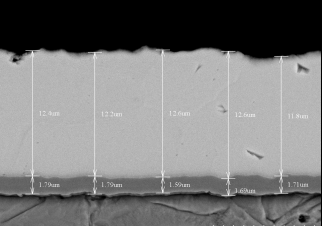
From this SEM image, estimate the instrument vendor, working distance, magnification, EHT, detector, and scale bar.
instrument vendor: Hitachi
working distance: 8.7 mm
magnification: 4 K X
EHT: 15 kV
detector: BSE3D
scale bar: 10000 nm